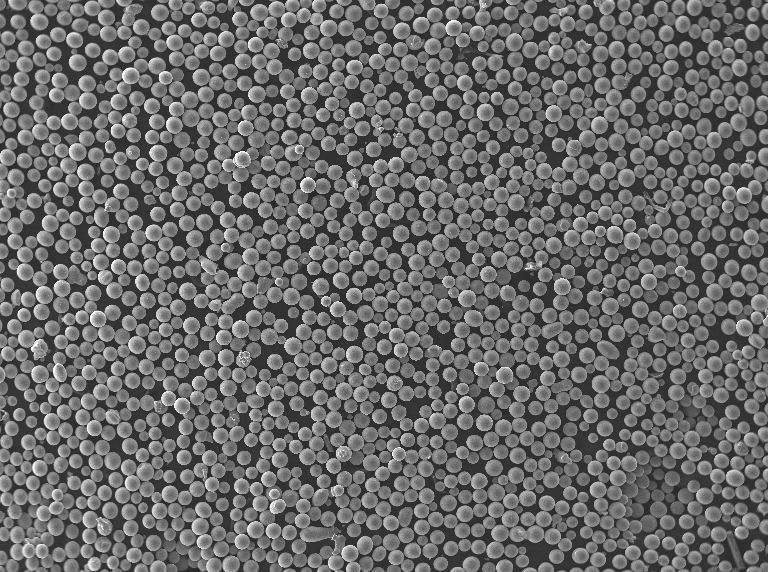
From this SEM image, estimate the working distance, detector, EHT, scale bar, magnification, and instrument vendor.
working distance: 5.47 mm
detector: SE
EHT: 30 kV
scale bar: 500000 nm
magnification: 0.1 K X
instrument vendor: Tescan